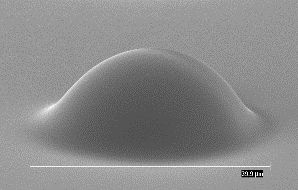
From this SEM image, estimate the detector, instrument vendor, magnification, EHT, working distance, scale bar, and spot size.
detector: SE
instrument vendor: FEI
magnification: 2.5 K X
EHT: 10 kV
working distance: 15.4 mm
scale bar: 10000 nm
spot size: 3